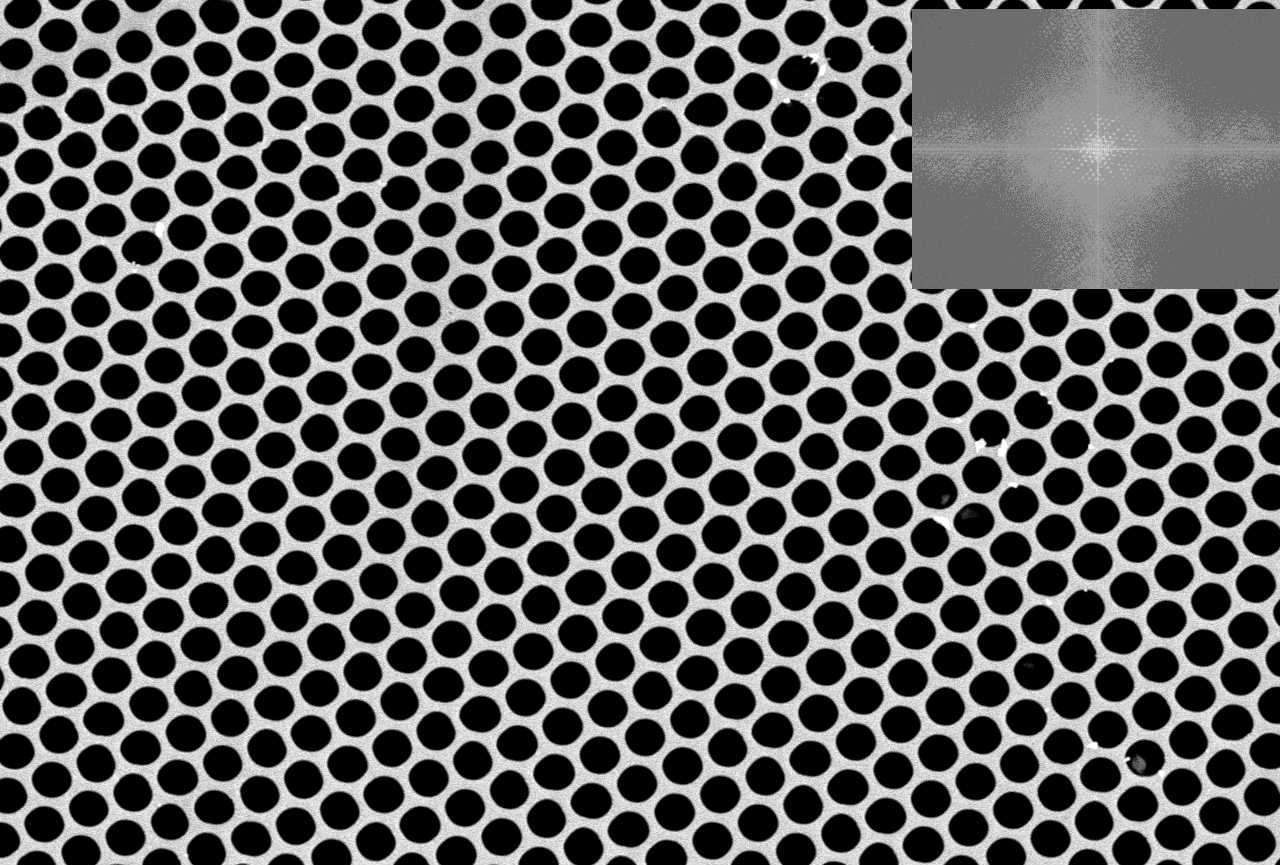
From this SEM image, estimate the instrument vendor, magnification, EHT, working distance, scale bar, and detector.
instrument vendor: Hitachi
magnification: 1 K X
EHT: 20 kV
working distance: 13.8 mm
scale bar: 50000 nm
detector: SE